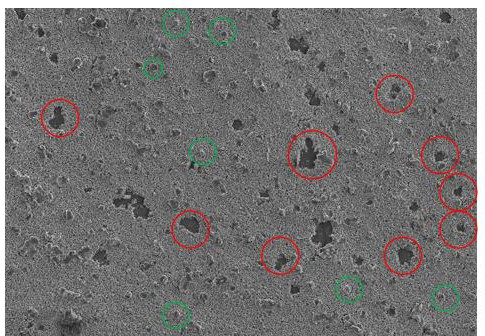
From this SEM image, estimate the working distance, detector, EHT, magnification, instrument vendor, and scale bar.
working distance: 10.6 mm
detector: SE(L)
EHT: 5 kV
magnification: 0.5 K X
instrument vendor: Hitachi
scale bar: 100000 nm